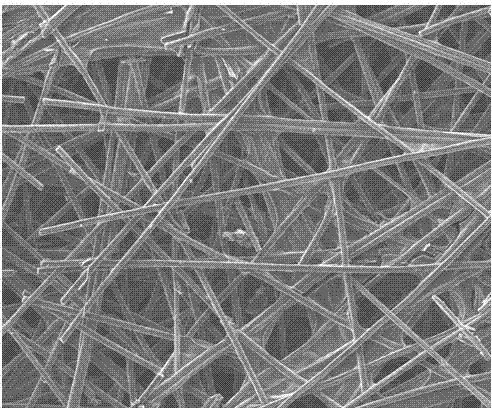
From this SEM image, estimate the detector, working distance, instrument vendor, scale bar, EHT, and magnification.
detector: LEI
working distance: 7.9 mm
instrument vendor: JEOL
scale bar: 100000 nm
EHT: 10 kV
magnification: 0.2 K X